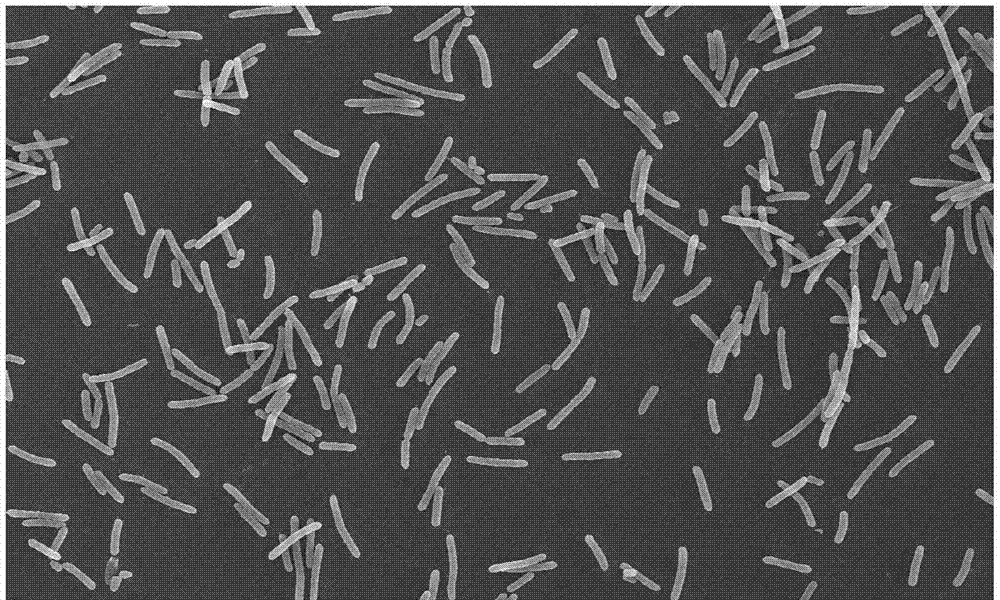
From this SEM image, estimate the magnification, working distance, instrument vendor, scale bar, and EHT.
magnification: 2 K X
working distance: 8.1 mm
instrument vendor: Hitachi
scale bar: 20000 nm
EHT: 10 kV